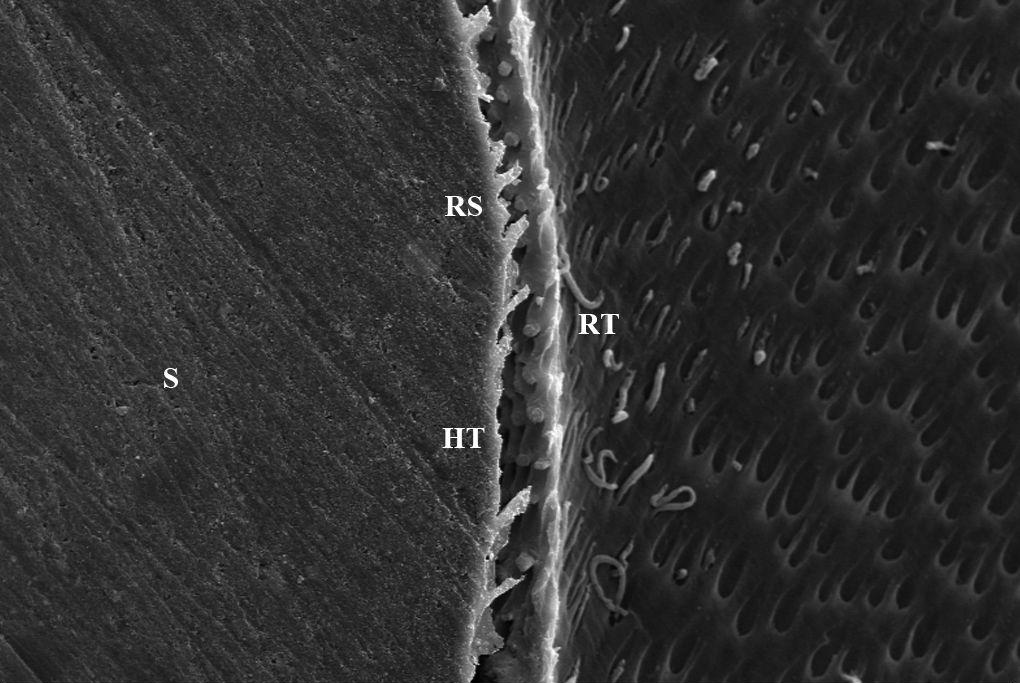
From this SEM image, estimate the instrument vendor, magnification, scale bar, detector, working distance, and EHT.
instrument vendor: Zeiss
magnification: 0.5 K X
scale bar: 10000 nm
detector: SE1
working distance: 11 mm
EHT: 20 kV